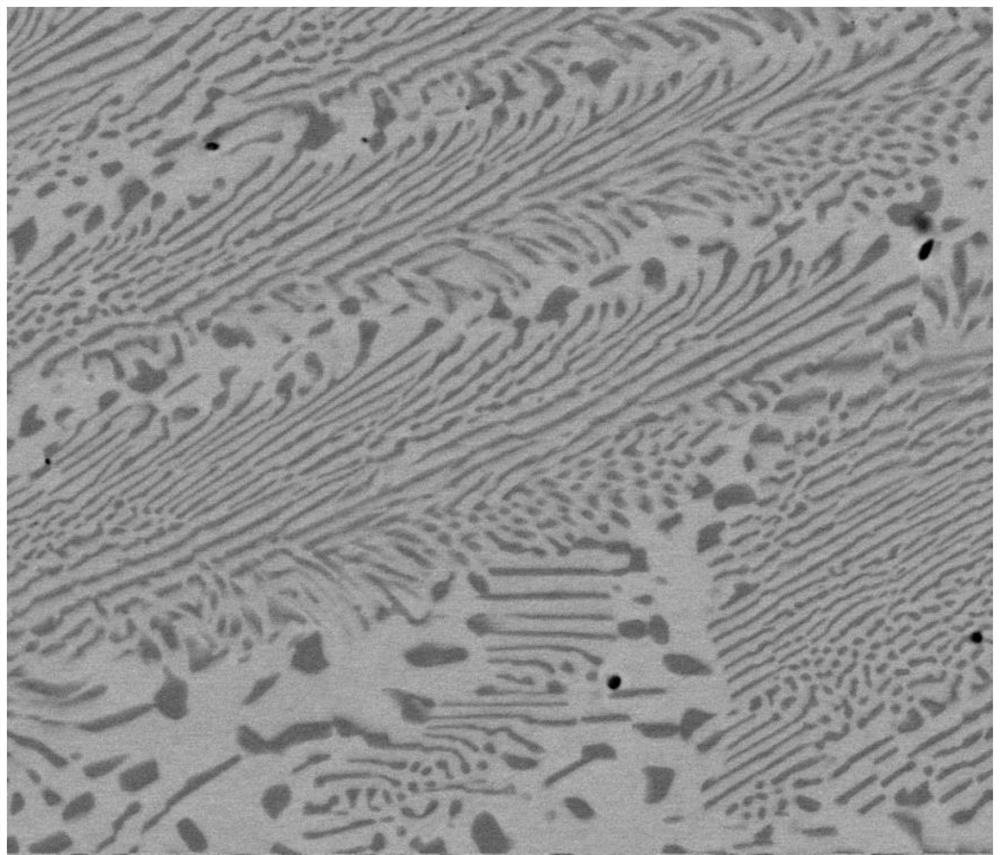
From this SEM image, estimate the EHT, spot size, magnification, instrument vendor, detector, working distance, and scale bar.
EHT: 20 kV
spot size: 5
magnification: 5 K X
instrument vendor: FEI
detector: vCD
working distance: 13.9 mm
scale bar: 20000 nm